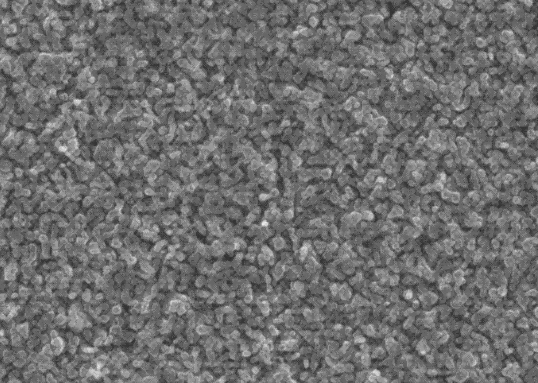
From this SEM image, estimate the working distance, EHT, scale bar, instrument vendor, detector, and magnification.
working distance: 9.8 mm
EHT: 20 kV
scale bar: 1000 nm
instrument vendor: FEI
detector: ETD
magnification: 100 K X